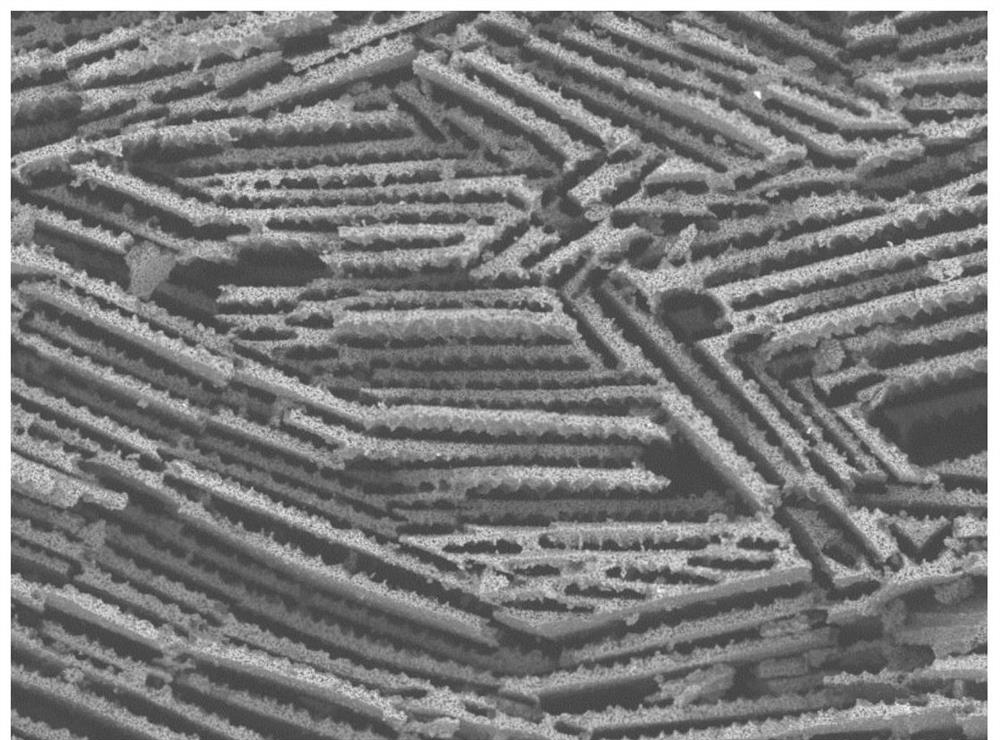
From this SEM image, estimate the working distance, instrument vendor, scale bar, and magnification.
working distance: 6.6 mm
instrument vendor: Hitachi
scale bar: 300000 nm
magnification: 0.3 K X